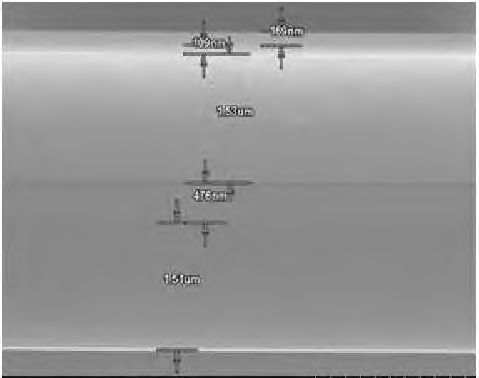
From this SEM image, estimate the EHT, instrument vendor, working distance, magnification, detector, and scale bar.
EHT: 15 kV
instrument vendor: Hitachi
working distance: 10.4 mm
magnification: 20 K X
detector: SE(M)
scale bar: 2000 nm